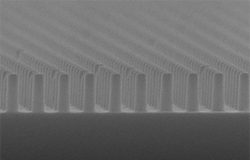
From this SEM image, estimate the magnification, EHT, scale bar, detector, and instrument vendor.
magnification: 30 K X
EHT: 20 kV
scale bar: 500 nm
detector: SEI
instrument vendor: JEOL